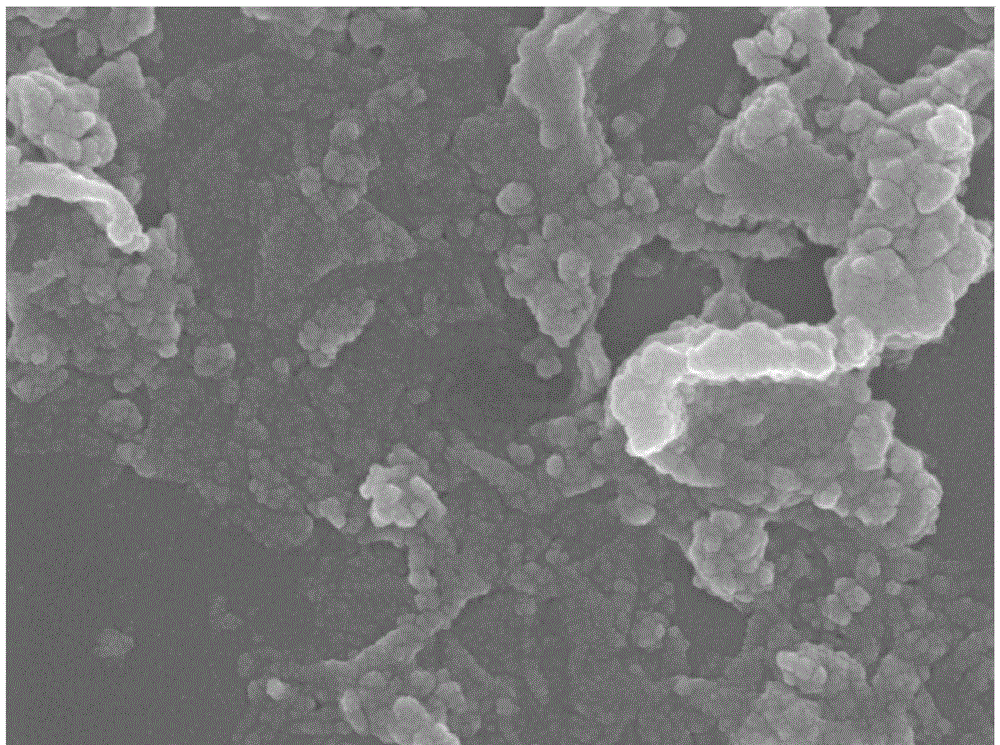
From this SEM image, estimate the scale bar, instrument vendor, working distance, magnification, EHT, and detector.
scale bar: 100 nm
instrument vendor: JEOL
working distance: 7 mm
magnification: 50 K X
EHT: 5 kV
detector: SEI